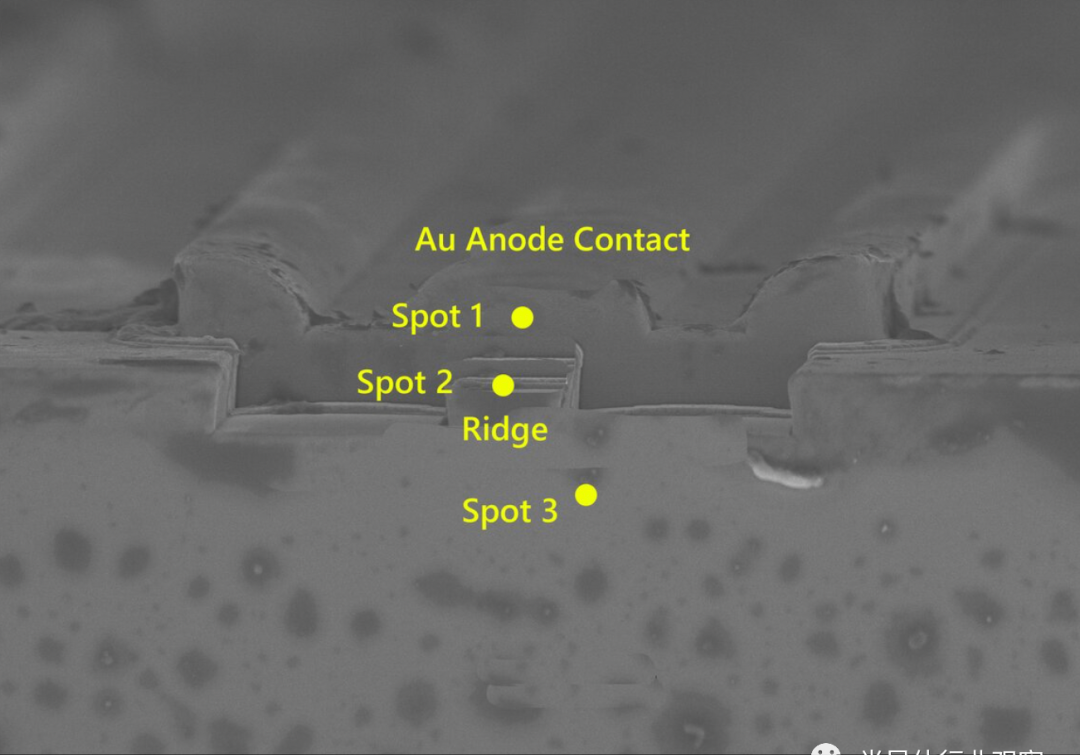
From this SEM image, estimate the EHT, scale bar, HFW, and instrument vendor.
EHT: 5 kV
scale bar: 2000 nm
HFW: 36.47 µm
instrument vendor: Zeiss